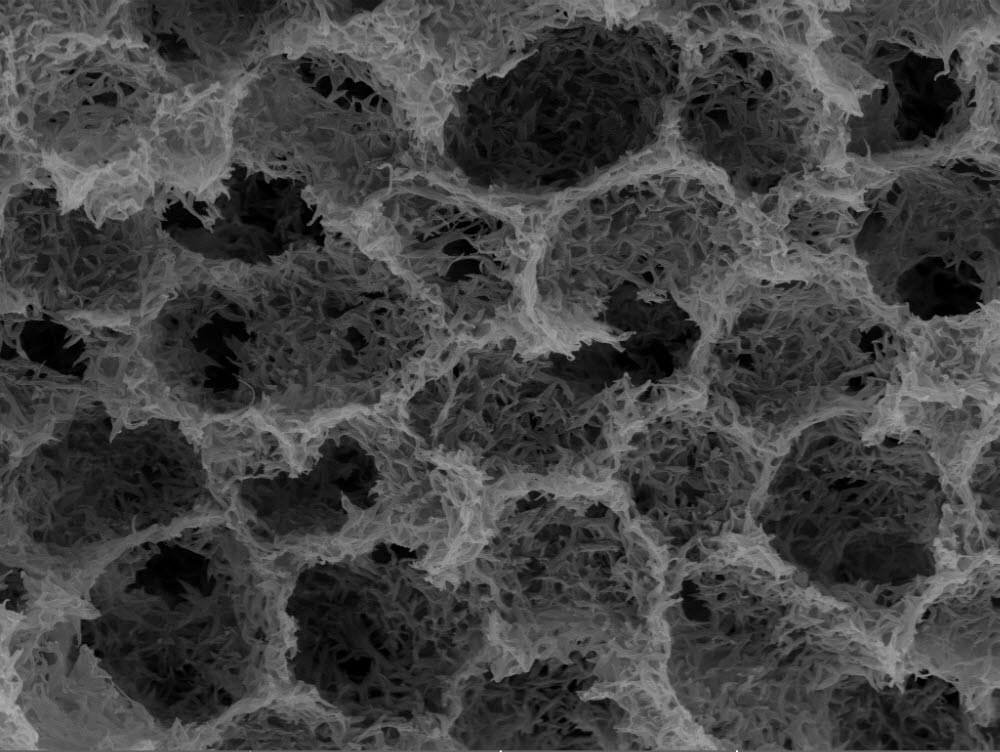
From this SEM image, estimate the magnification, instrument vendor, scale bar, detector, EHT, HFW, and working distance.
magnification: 3 K X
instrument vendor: Tescan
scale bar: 20000 nm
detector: In-Beam SE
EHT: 10 kV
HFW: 84.8 µm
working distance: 4.2 mm